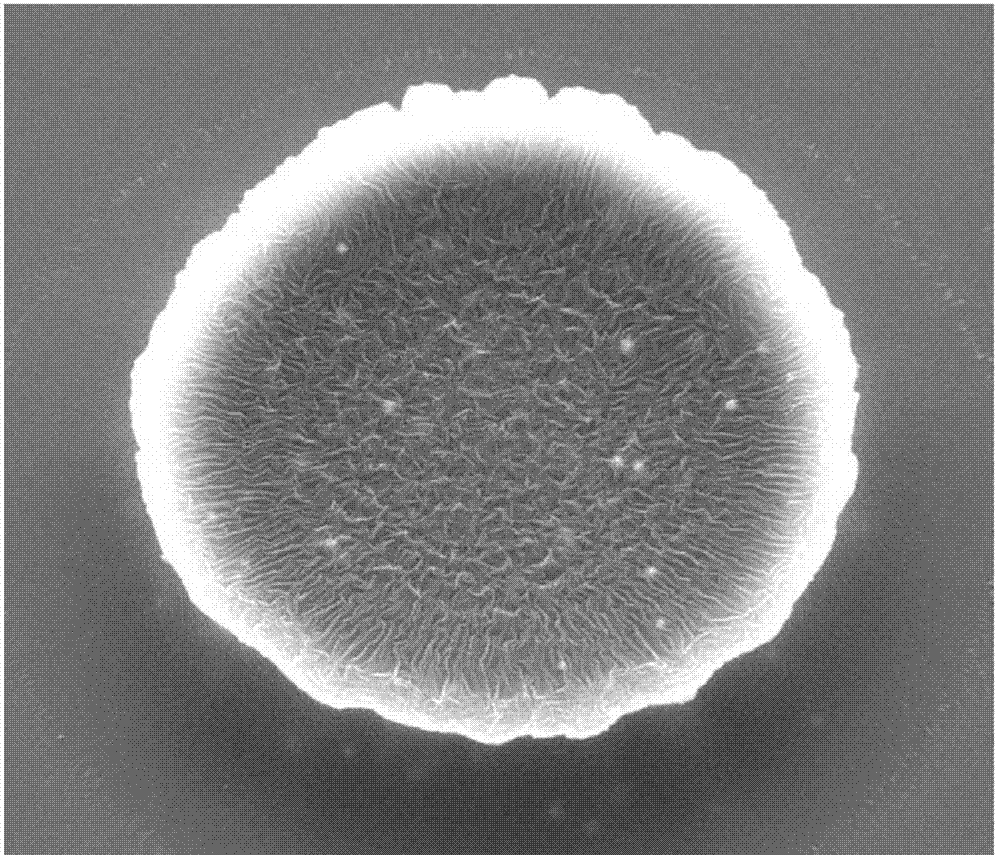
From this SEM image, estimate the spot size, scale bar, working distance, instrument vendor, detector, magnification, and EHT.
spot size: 3.5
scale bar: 20000 nm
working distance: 18.1 mm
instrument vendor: FEI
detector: ETD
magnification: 2 K X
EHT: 20 kV